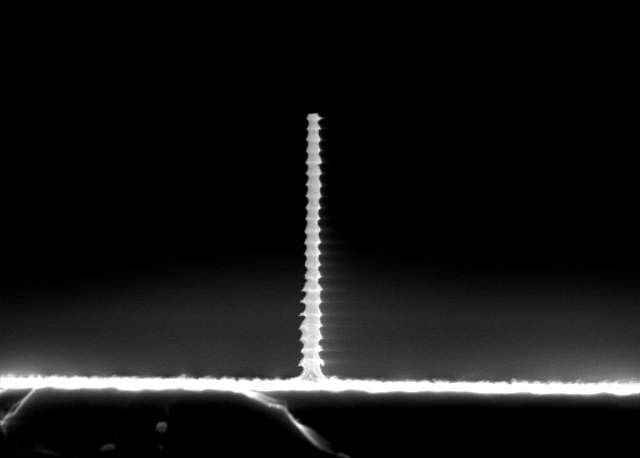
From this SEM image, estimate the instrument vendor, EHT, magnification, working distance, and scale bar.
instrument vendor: JEOL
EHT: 15 kV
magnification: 5 K X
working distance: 7 mm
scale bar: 5000 nm